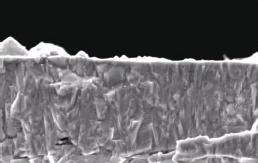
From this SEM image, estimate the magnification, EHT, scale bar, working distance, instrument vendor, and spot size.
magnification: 15 K X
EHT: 5 kV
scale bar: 2000 nm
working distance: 10.1 mm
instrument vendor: FEI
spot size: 3